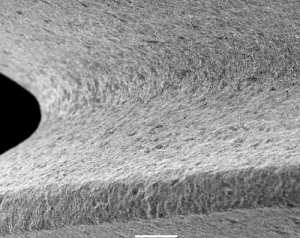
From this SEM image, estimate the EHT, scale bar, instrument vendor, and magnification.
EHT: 15 kV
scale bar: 100000 nm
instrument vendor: JEOL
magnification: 0.15 K X